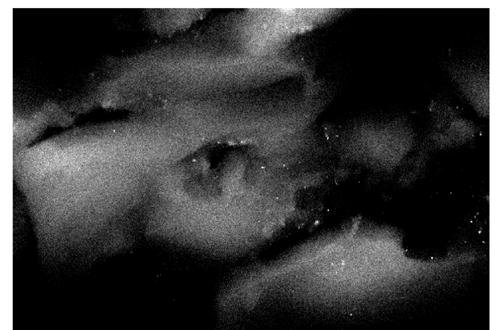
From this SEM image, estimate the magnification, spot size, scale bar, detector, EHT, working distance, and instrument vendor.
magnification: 10 K X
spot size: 4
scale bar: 5000 nm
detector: SE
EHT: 25 kV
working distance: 4.9 mm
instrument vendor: FEI